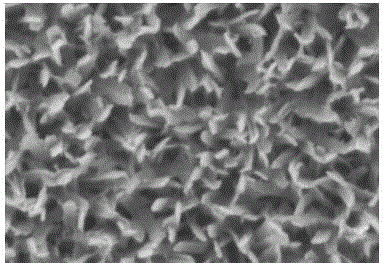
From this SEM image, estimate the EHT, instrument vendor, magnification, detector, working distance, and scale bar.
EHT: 5 kV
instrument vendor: Hitachi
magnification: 100 K X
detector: SE(M)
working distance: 5.2 mm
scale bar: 500 nm